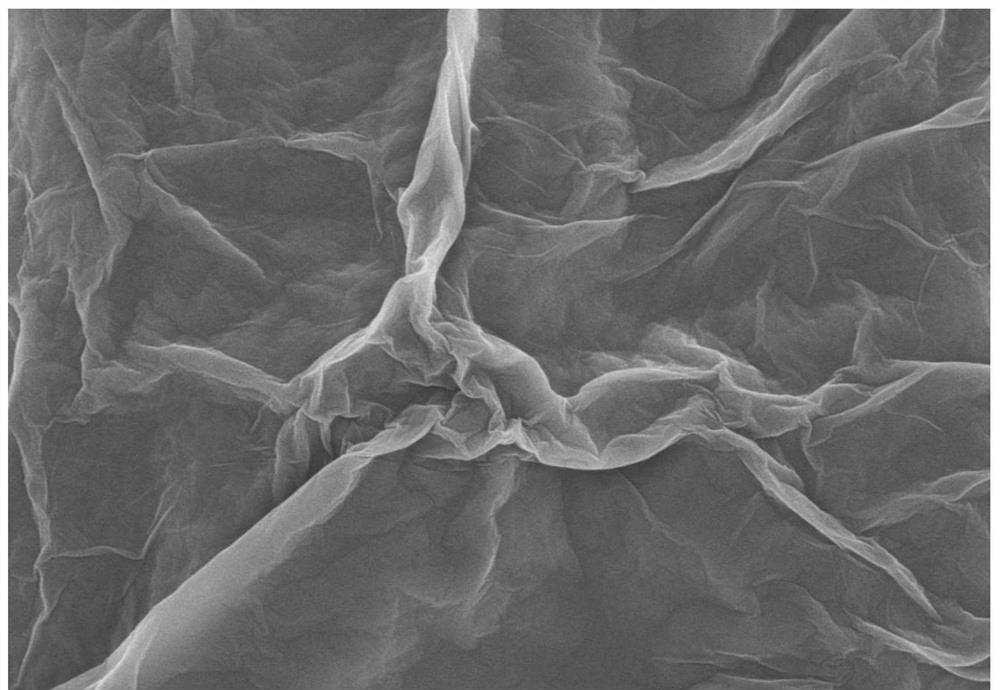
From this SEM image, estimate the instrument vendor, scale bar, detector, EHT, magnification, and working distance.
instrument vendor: Hitachi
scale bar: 2000 nm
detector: SE(UL)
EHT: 5 kV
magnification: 20 K X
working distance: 8.4 mm